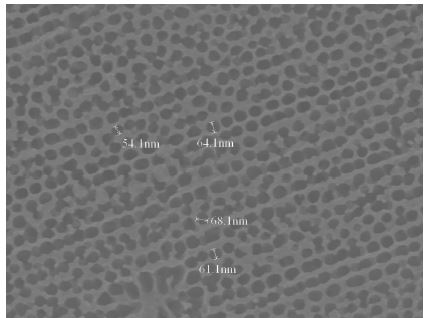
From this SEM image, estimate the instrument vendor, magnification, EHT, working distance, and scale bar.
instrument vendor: JEOL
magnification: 50 K X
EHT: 10 kV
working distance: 6.4 mm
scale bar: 100 nm